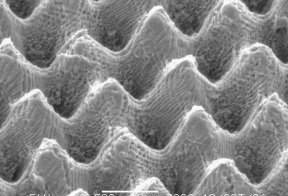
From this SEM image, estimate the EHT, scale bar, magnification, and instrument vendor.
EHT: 5 kV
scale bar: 10000 nm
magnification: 2.5 K X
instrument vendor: JEOL